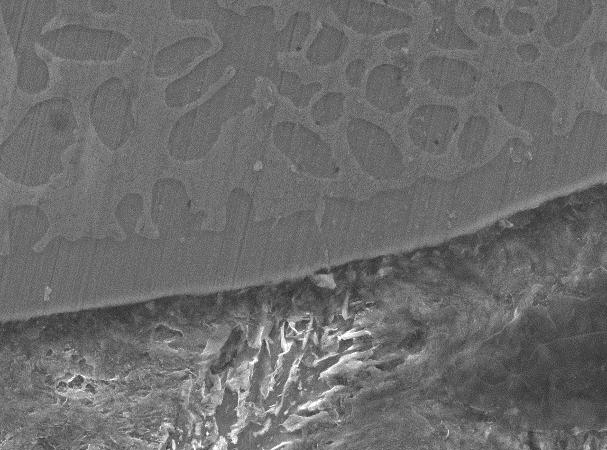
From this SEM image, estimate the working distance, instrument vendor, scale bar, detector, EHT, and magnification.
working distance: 10.2 mm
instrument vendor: JEOL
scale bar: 10000 nm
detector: SEI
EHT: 20 kV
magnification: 2 K X